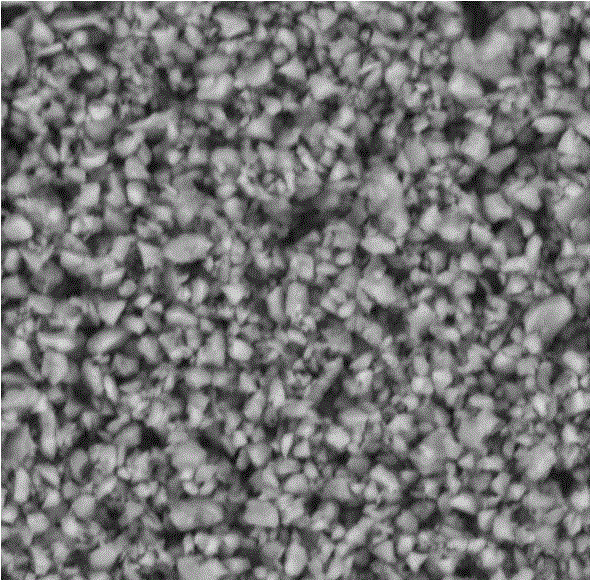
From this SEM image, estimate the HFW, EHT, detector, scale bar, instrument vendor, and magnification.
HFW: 16.1 µm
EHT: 10 kV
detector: BSD Full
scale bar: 5000 nm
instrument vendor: other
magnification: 16.5 K X